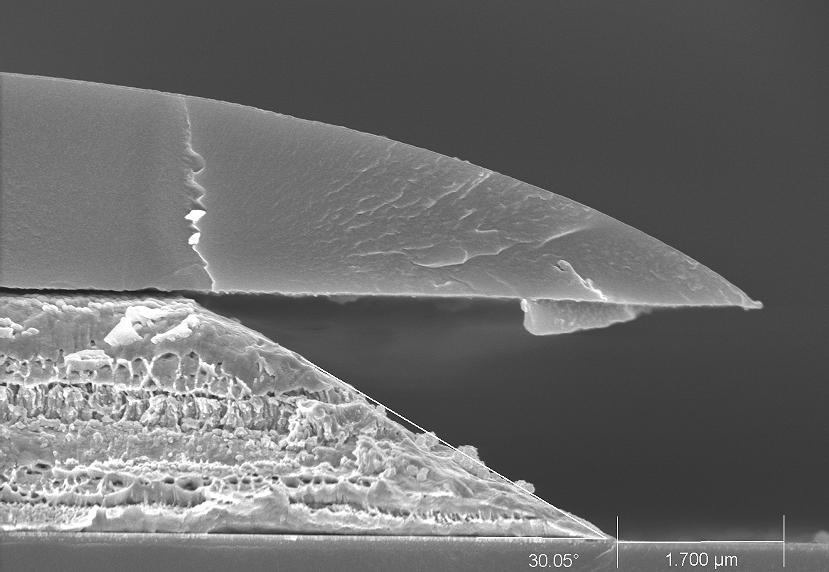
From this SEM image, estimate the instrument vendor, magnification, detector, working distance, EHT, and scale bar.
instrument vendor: Hitachi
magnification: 15 K X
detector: SE(U)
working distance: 9 mm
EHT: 5 kV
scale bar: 3000 nm